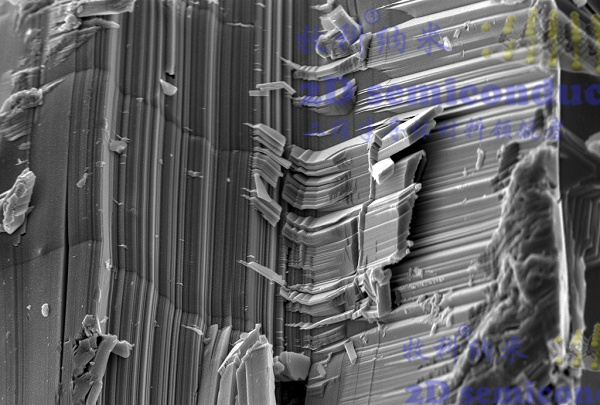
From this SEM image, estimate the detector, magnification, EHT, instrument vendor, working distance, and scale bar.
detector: InLens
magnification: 10 K X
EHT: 5 kV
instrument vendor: Zeiss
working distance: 5.1 mm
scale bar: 1000 nm